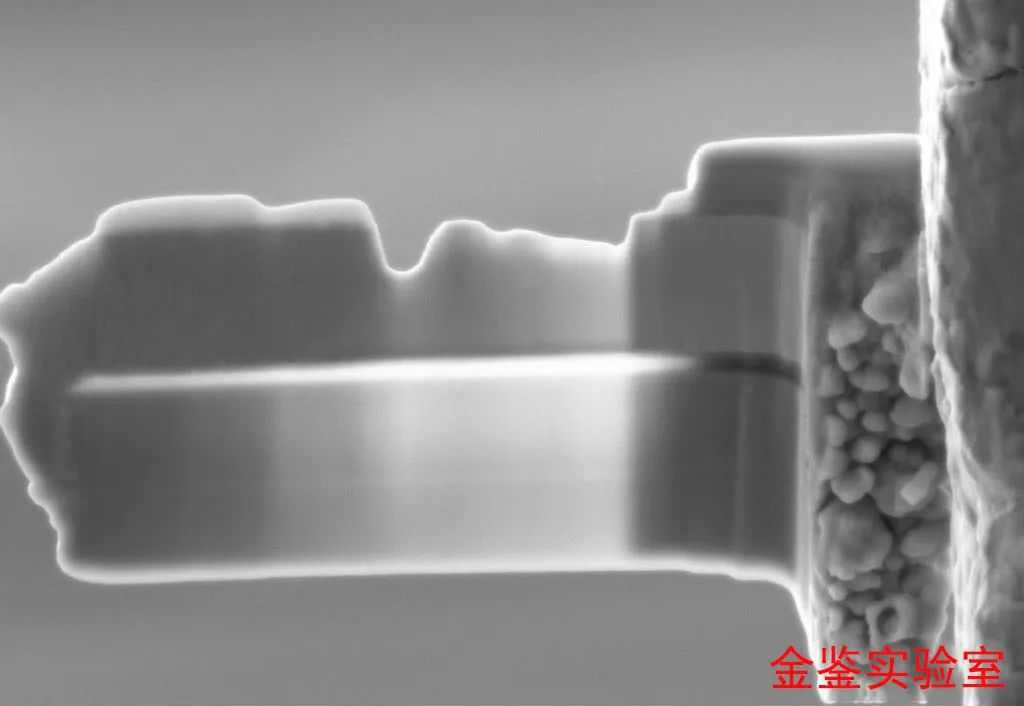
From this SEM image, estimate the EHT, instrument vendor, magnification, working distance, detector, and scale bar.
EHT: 5 kV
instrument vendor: Zeiss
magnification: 17.62 K X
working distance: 5.2 mm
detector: SE2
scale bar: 200 nm